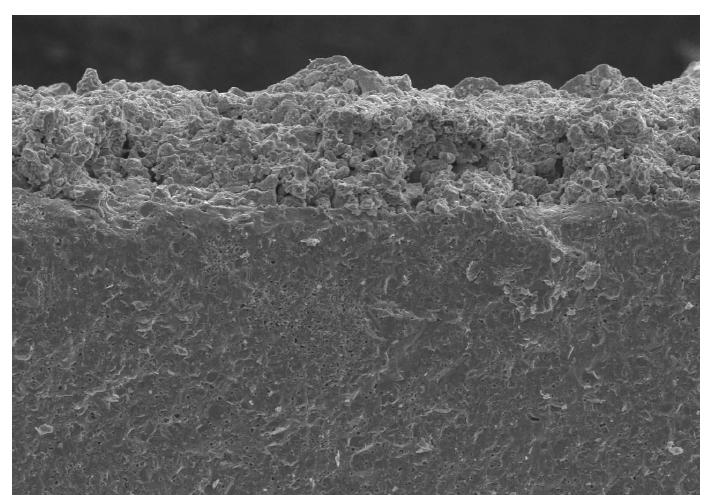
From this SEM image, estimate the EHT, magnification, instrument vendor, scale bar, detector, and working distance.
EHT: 15 kV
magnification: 0.5 K X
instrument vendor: Hitachi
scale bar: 100000 nm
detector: SE(U)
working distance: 12.7 mm